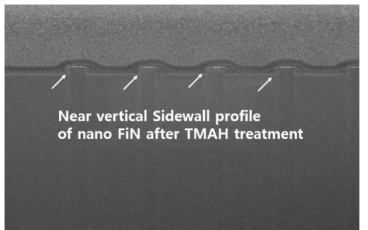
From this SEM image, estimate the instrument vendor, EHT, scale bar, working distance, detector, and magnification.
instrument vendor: FEI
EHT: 2 kV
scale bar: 1000 nm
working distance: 4.2 mm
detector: TLD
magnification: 40 K X